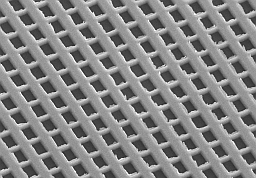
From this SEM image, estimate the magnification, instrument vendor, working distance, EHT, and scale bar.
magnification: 10 K X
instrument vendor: Hitachi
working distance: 12.3 mm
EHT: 10 kV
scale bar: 5000 nm